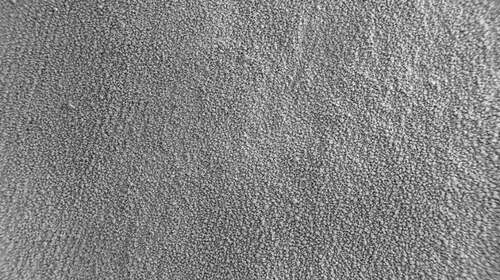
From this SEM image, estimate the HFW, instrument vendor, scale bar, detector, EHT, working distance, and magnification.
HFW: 3851 µm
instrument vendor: Zeiss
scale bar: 300000 nm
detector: SE2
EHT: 3 kV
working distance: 9.3 mm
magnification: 0.03 K X